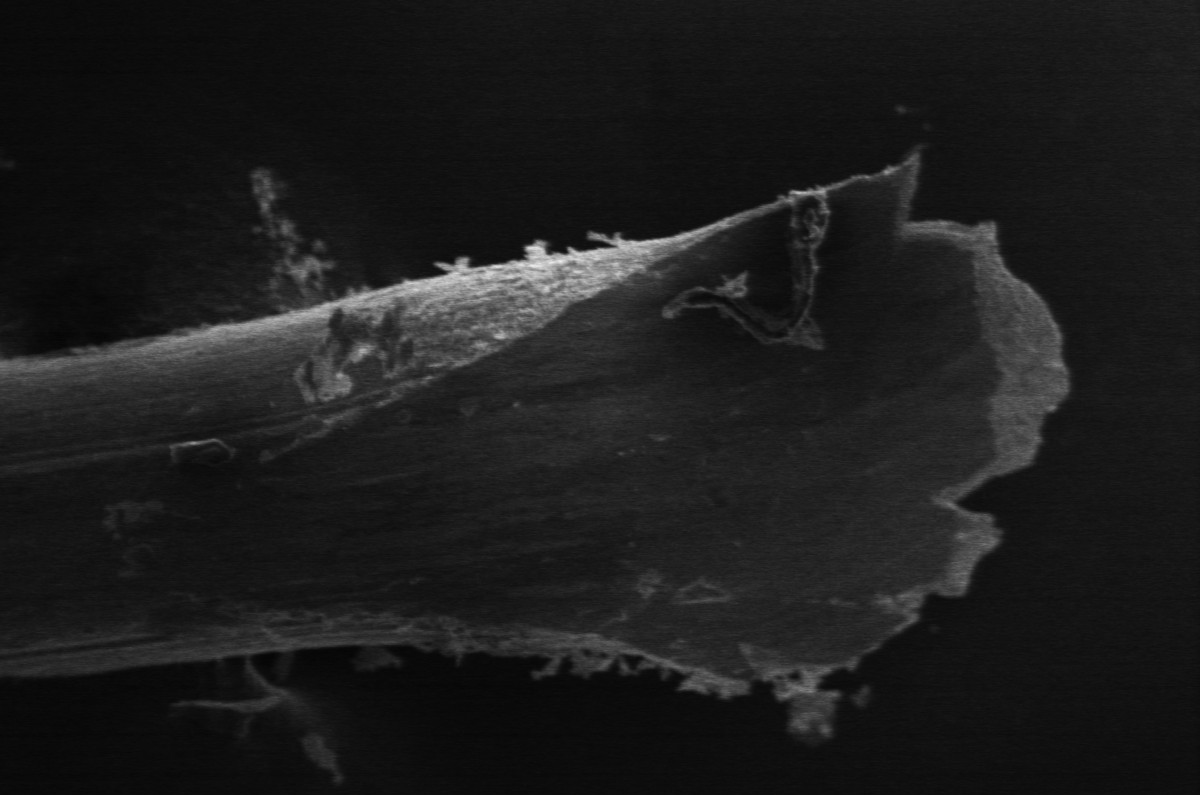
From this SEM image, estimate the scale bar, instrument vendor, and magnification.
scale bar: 100000 nm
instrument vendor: Hitachi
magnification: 0.35 K X